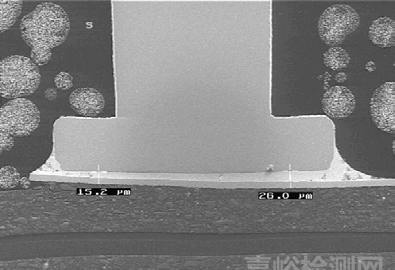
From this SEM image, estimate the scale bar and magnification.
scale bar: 200000 nm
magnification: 0.1 K X